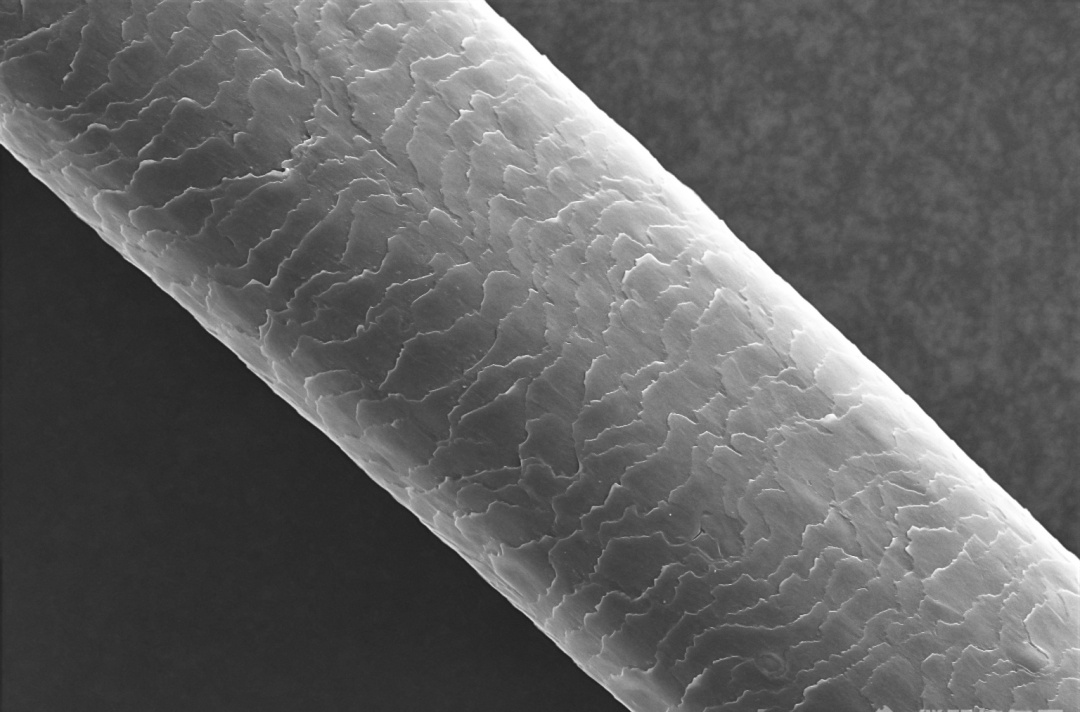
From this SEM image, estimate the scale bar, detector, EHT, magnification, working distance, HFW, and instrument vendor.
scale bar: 50000 nm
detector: ETD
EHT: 15 kV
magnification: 0.5 K X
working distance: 8 mm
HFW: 254 µm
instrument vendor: Thermo